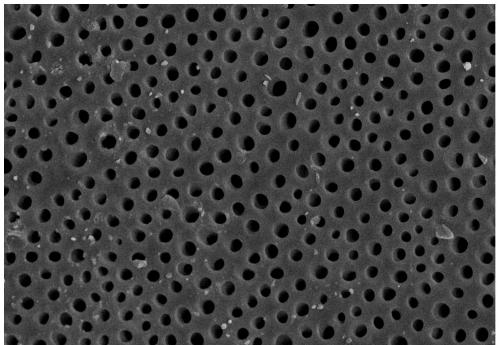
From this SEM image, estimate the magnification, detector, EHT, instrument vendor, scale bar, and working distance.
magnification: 1 K X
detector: SE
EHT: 15 kV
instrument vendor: Hitachi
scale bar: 50000 nm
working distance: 9 mm